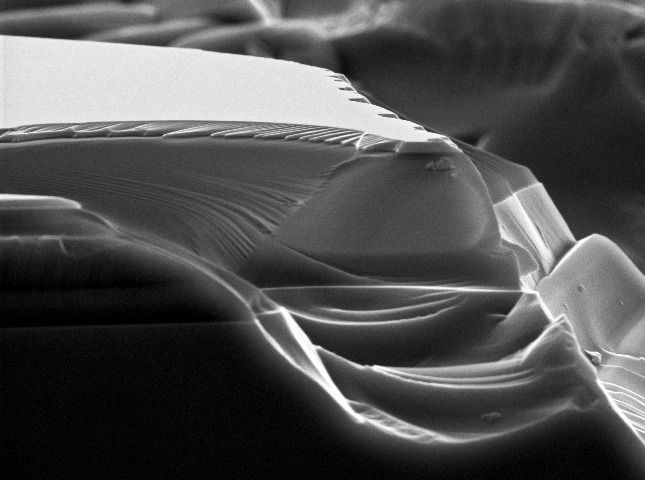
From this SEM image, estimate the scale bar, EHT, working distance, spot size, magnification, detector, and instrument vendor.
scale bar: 2000 nm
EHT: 10 kV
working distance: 15.6 mm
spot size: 3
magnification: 7.54 K X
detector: SE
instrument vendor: FEI